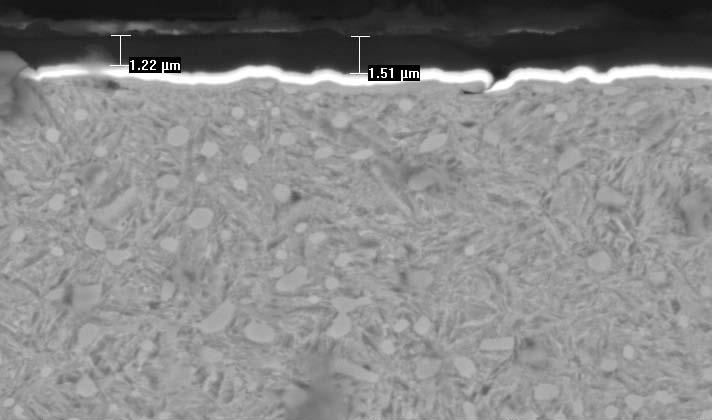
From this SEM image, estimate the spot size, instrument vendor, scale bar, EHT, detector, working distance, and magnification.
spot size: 3.7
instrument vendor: FEI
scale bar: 5000 nm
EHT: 20 kV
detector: BSE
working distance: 10.9 mm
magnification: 3.5 K X